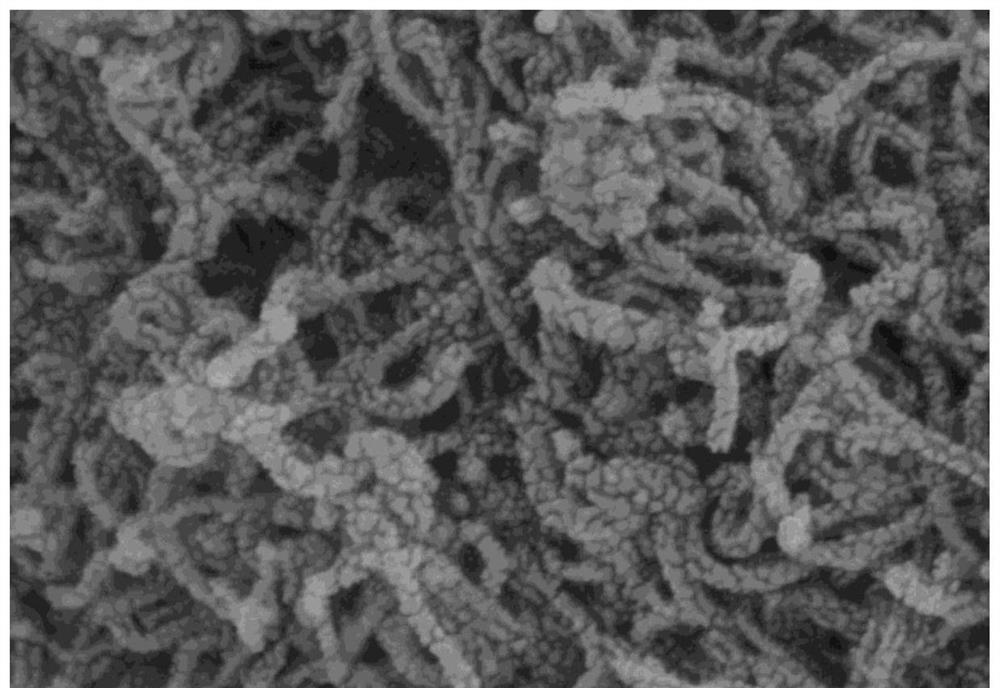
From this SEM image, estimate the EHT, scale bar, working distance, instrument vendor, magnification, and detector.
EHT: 5 kV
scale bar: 500 nm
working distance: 7.8 mm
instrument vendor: Hitachi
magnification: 100 K X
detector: SE(UL)